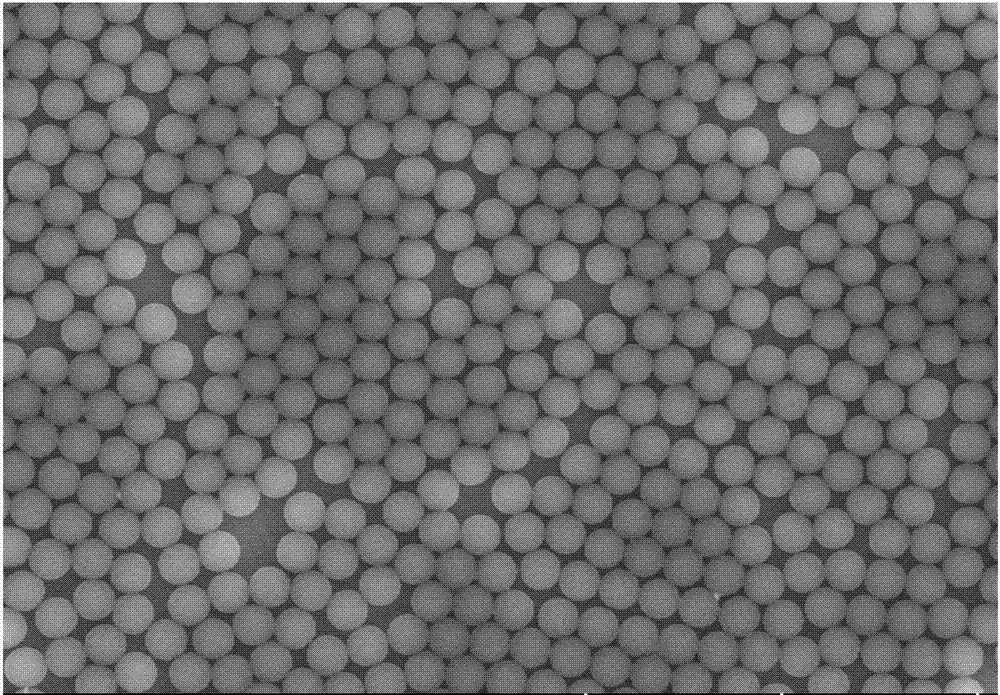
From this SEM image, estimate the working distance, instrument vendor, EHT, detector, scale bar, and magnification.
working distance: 13.5 mm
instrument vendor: Hitachi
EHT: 10 kV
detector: SE(UL)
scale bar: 10000 nm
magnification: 5 K X